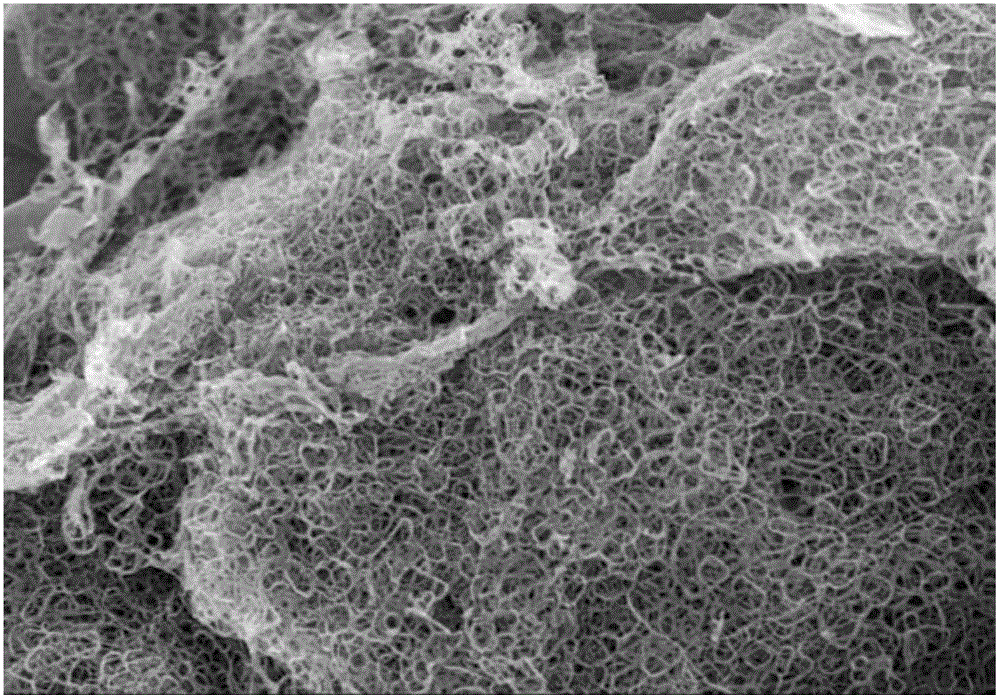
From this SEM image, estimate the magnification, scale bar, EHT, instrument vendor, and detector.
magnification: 10 K X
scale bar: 5000 nm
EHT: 5 kV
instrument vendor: Hitachi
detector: SE(M)